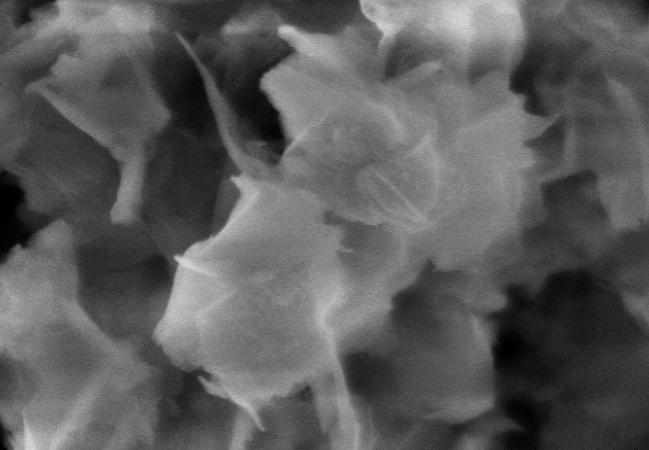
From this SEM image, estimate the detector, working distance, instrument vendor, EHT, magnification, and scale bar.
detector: SE(M)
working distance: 8.2 mm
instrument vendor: Hitachi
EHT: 5 kV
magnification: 100 K X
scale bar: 500 nm